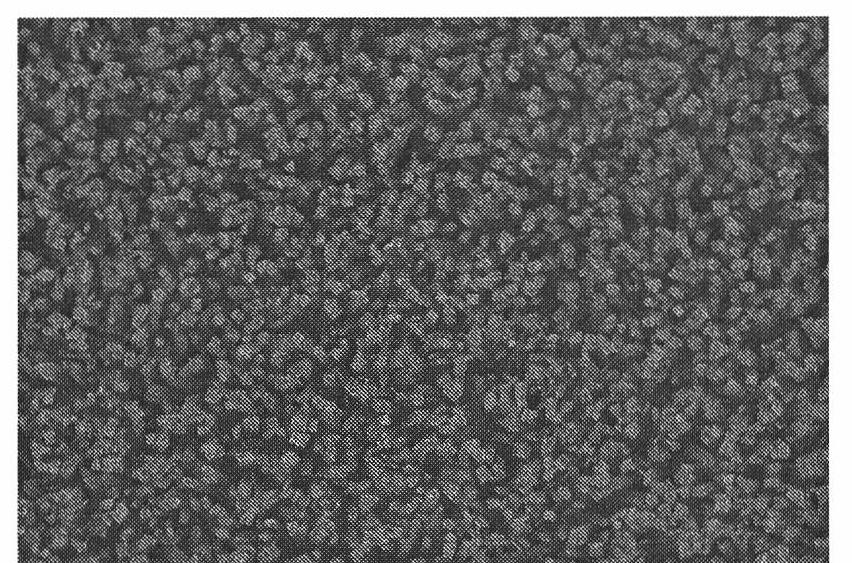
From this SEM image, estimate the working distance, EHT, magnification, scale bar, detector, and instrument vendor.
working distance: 5.1 mm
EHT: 15 kV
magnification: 10 K X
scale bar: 5000 nm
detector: SE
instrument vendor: Hitachi